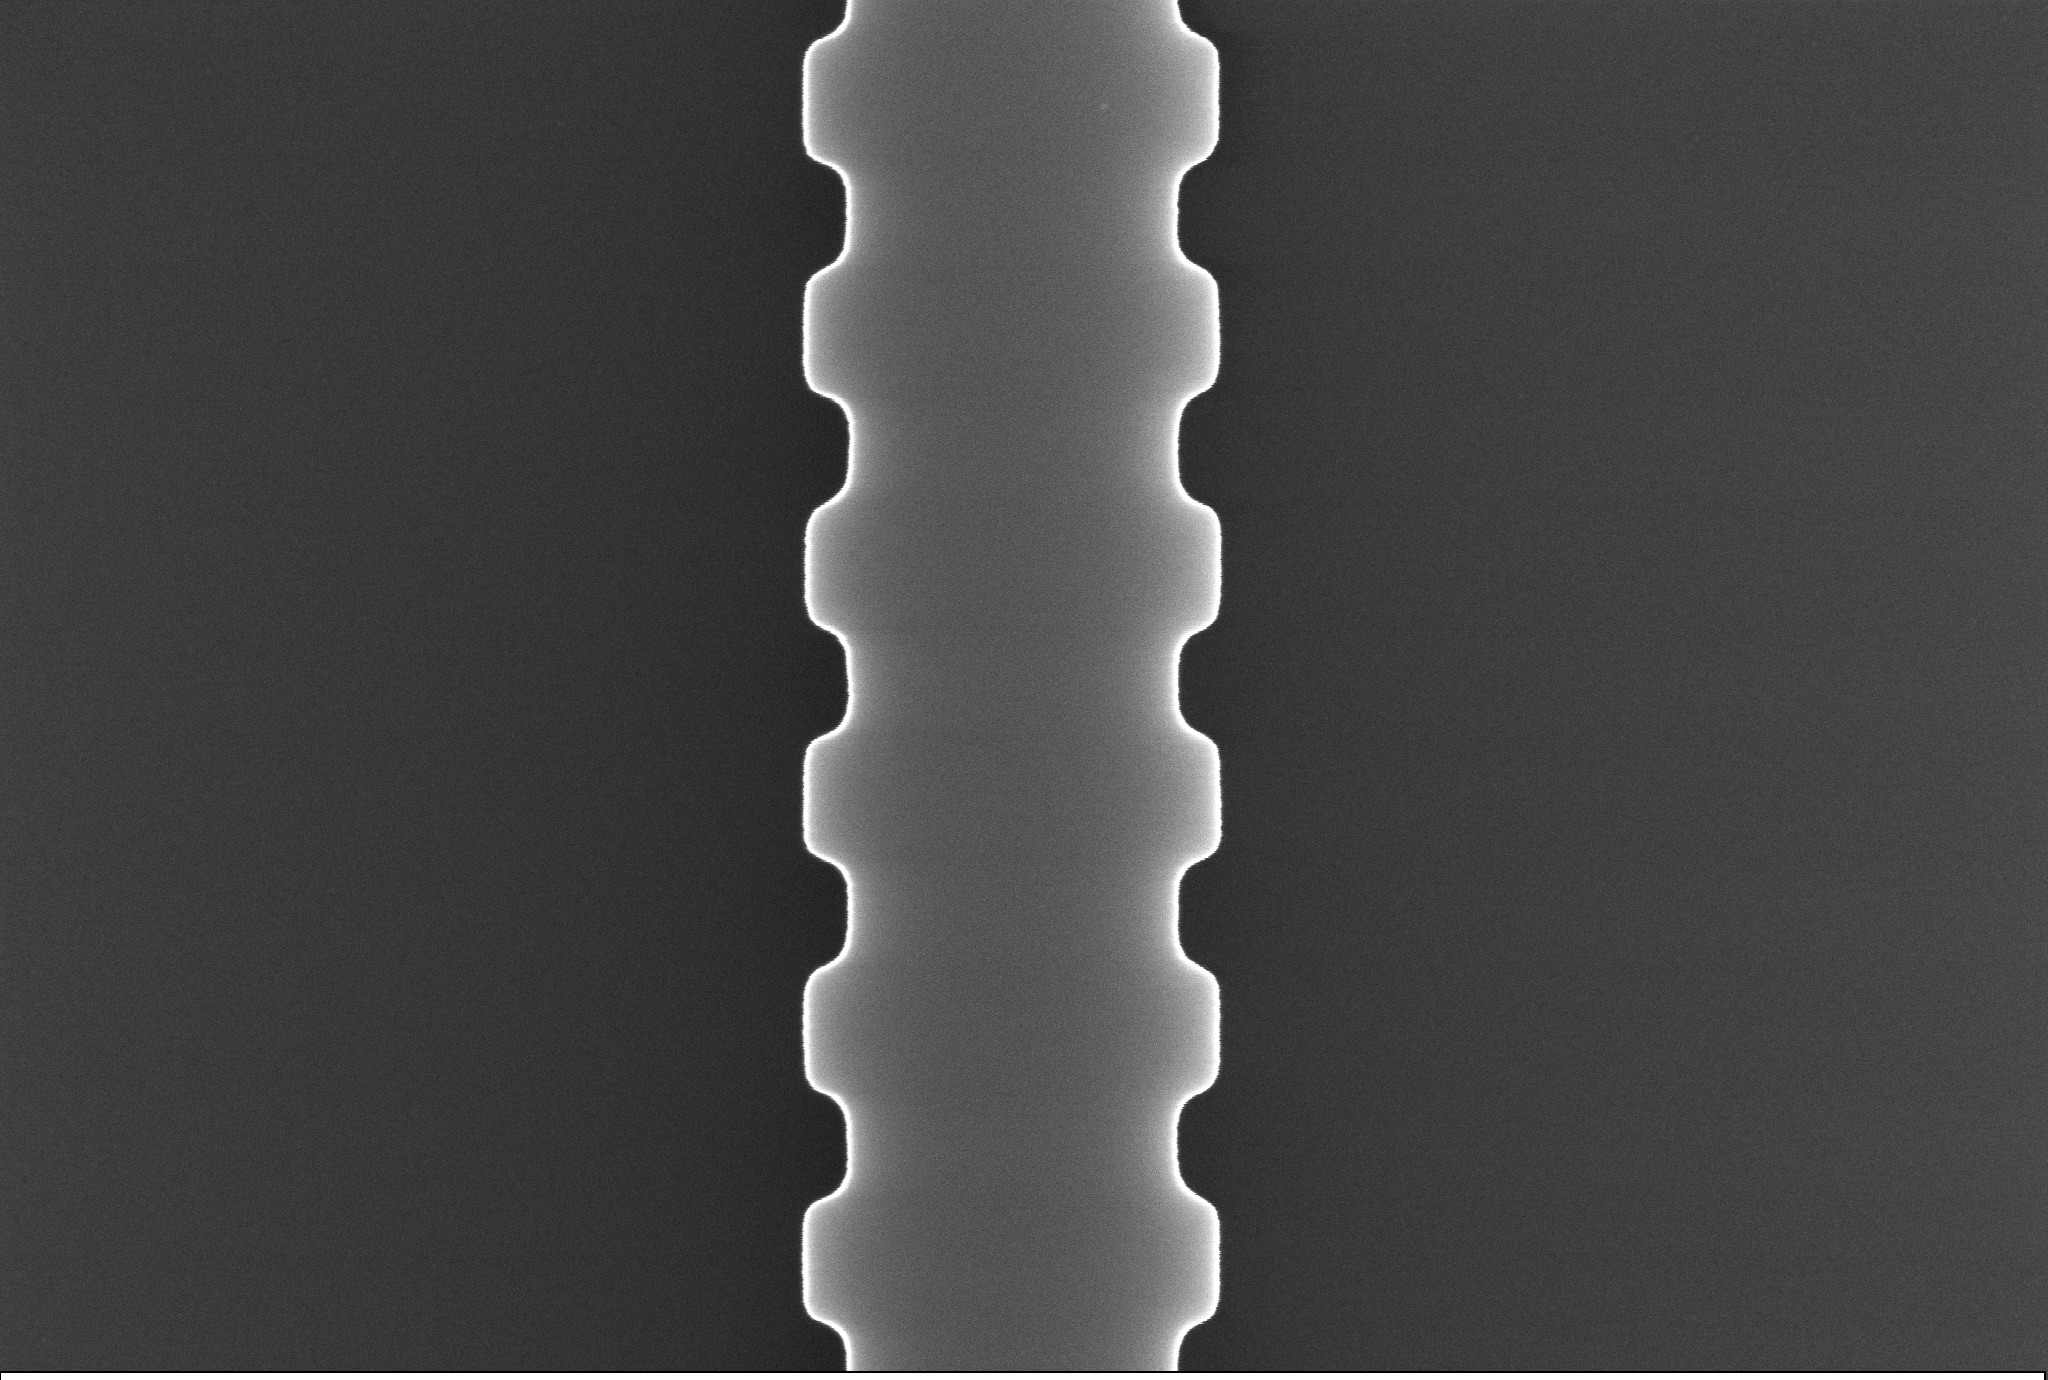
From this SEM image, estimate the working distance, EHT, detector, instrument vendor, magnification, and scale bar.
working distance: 6.7 mm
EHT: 15 kV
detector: InLens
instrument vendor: Zeiss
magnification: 40.82 K X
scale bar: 200 nm